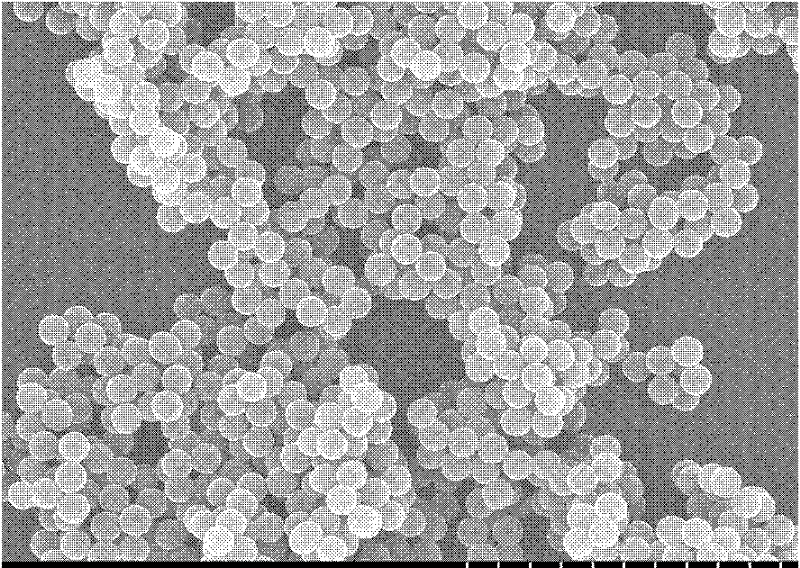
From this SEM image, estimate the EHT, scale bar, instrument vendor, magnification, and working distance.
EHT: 20 kV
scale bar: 5000 nm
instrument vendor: Hitachi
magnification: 10 K X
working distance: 12.8 mm